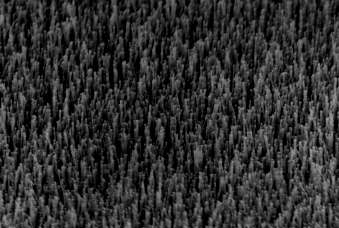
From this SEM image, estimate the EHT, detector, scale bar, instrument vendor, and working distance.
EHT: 15 kV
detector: InLens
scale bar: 1000 nm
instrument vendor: Zeiss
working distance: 5 mm